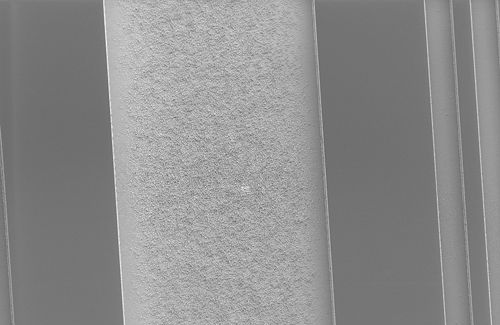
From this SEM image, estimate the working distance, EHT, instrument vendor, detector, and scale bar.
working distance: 11.7 mm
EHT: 2.5 kV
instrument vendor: Zeiss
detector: SE2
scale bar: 10000 nm